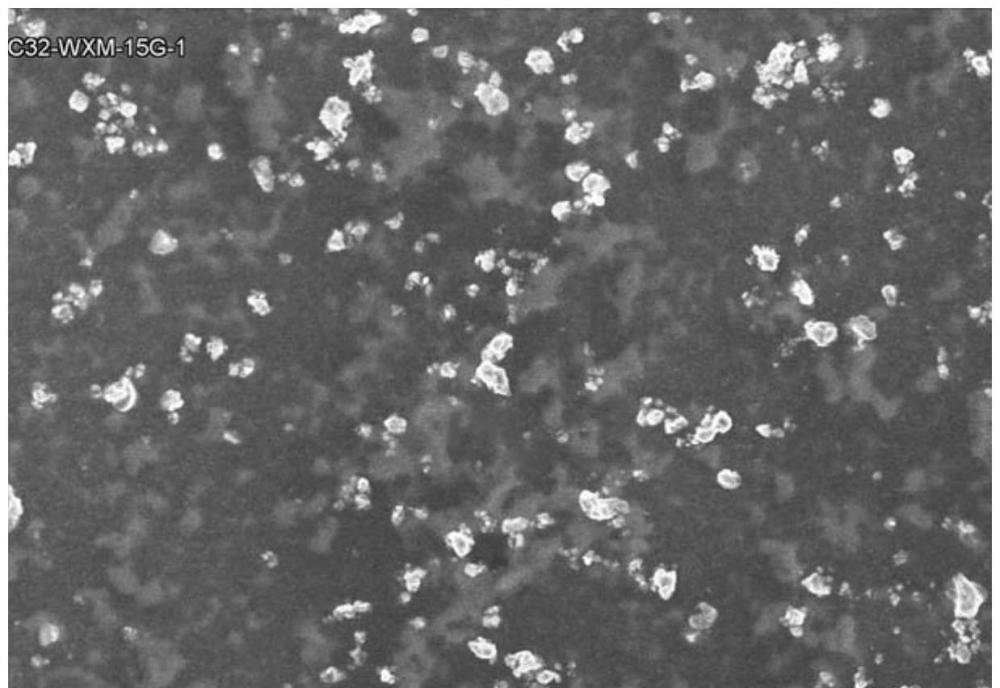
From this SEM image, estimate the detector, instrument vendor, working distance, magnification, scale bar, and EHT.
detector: SE(U)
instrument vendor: Hitachi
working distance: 8.3 mm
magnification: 1.5 K X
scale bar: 30000 nm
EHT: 5 kV